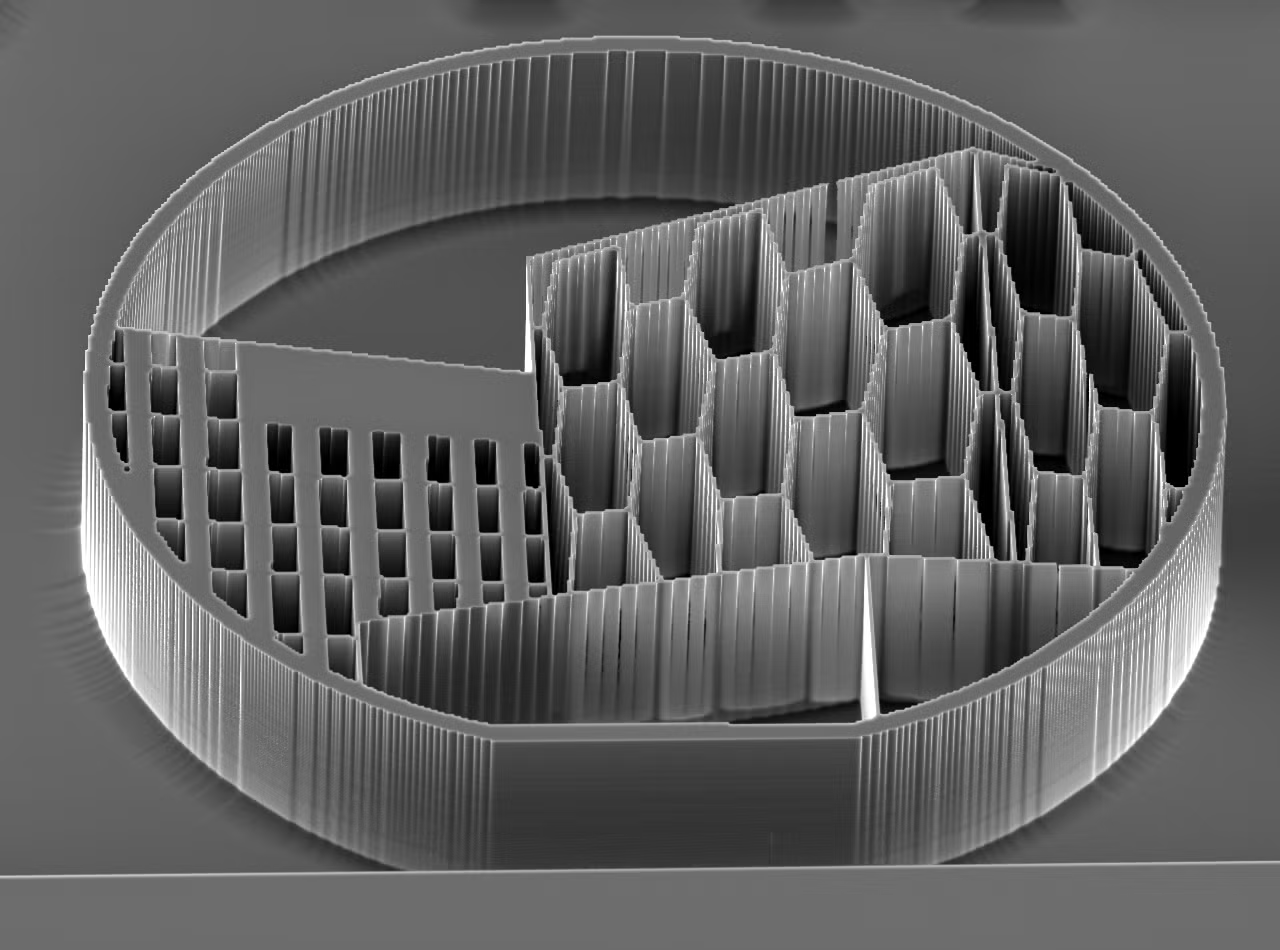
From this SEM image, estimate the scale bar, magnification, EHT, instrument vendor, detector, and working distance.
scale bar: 100000 nm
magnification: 0.15 K X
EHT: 20 kV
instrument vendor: Hitachi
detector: SEM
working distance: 15.2 mm